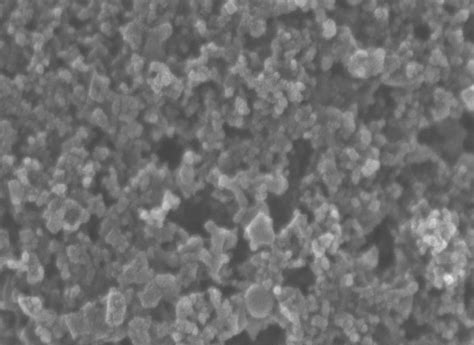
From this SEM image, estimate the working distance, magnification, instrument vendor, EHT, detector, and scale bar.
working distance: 4.7 mm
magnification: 100 K X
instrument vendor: Hitachi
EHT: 20 kV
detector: SE(U,LA0)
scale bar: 500 nm